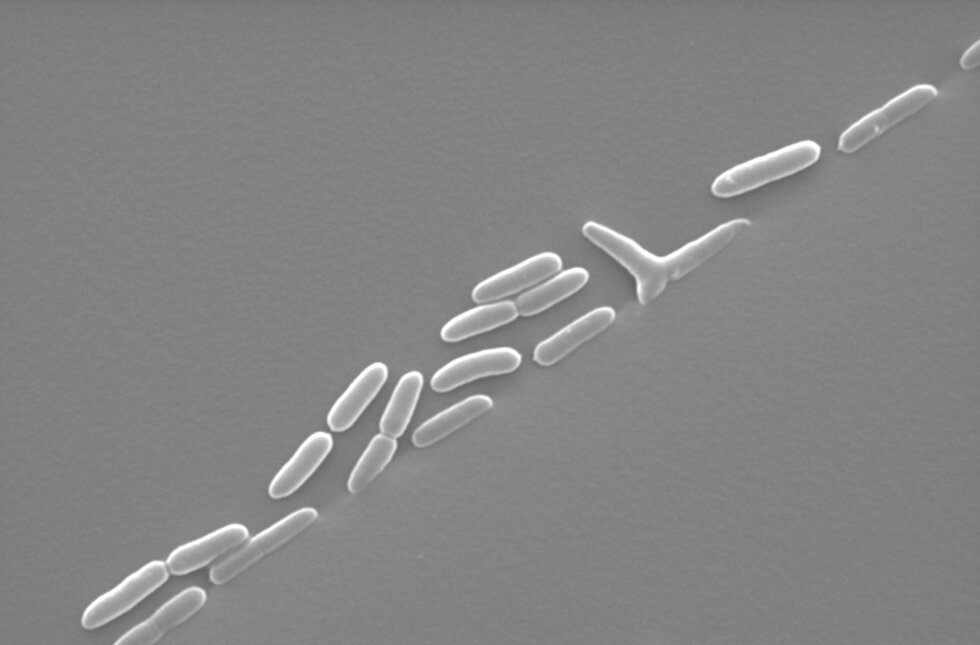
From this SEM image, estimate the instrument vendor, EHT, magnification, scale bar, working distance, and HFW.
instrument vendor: FEI/Thermo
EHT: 20 kV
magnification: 10 K X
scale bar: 5000 nm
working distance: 8.8 mm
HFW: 20.7 µm